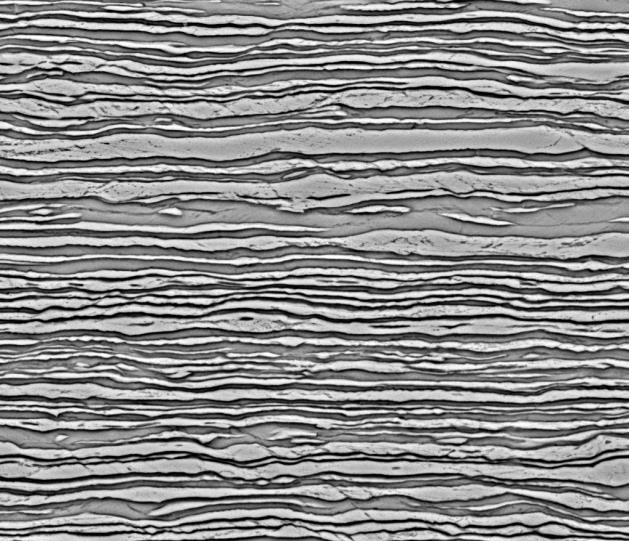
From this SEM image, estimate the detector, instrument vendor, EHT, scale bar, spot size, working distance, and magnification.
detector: BSED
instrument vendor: FEI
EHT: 20 kV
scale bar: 30000 nm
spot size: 3.5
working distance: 9.7 mm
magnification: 5 K X